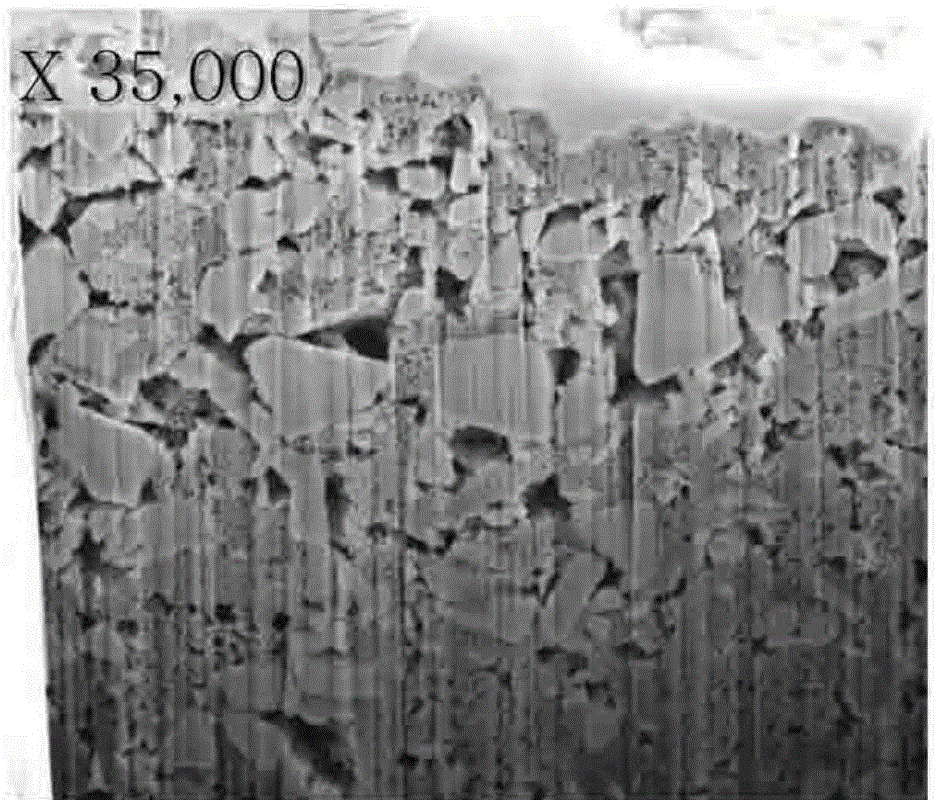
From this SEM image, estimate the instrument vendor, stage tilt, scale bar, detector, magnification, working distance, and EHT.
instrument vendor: FEI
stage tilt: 52°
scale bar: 3000 nm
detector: ETD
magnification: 35 K X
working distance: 9.9 mm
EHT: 5 kV